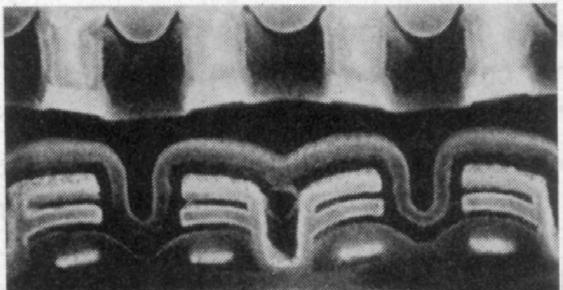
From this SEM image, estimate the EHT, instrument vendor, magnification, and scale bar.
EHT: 17 kV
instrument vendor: JEOL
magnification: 30.4 K X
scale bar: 329 nm